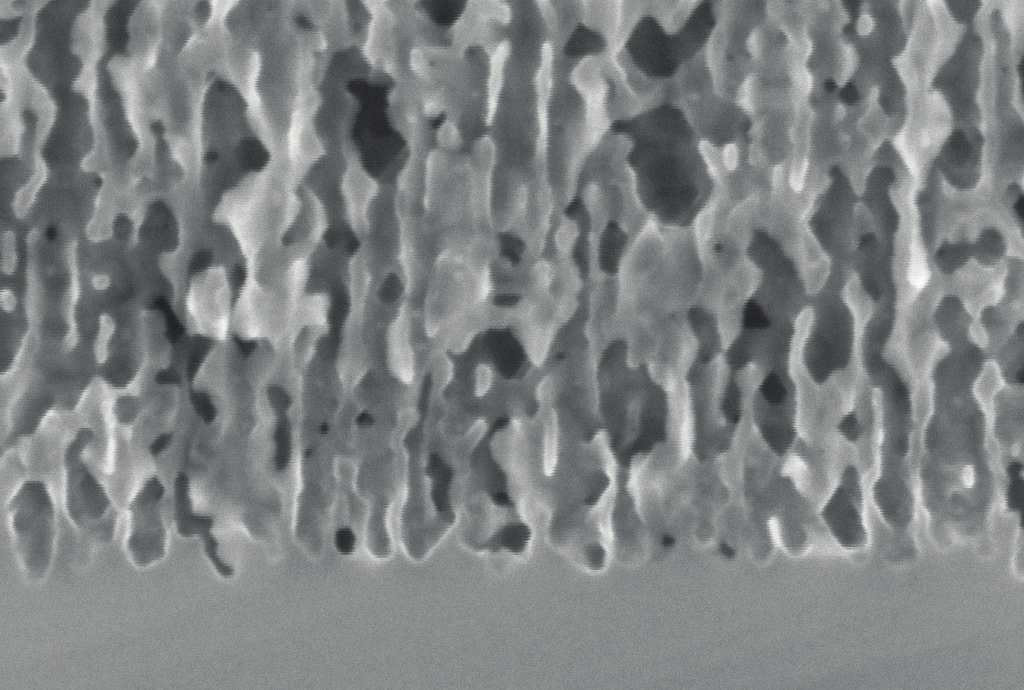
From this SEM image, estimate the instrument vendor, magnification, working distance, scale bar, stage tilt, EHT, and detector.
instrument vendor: Zeiss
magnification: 100 K X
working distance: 7.6 mm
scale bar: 100 nm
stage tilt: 0°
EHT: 5 kV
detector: InLens